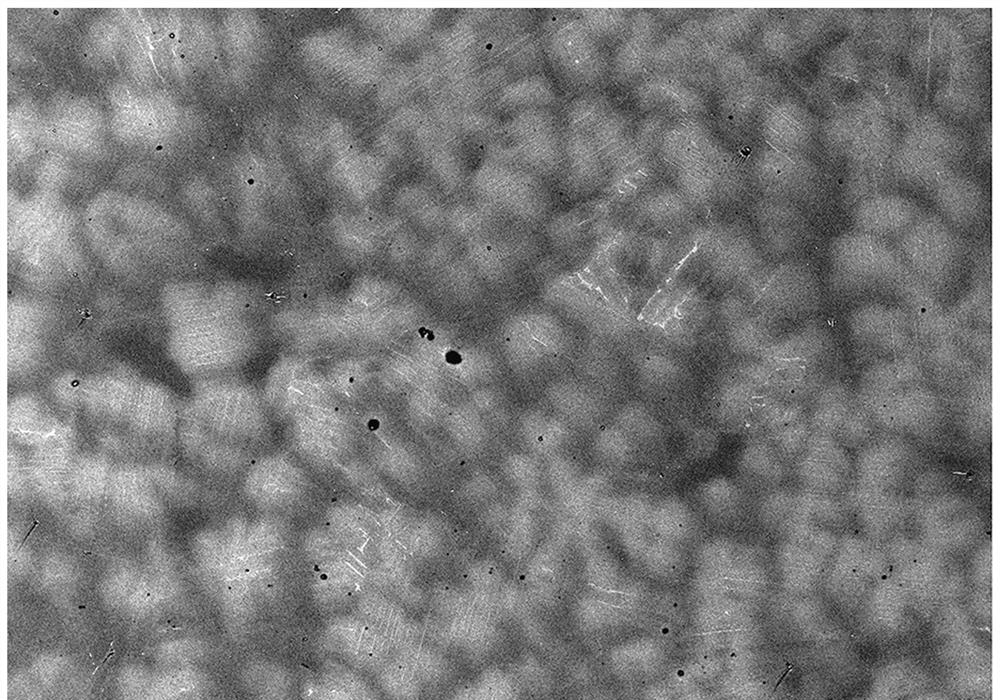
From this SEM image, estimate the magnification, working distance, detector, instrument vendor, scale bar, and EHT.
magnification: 0.15 K X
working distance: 6.1 mm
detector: BSE M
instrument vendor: Hitachi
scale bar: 300000 nm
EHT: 15 kV